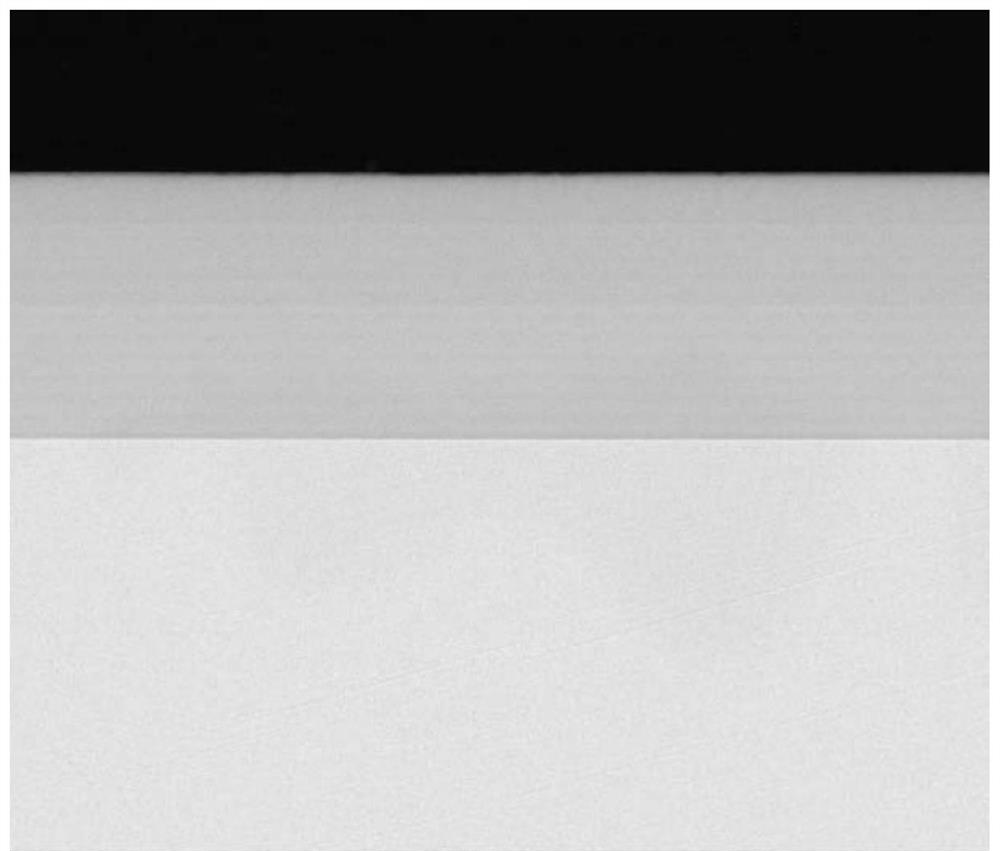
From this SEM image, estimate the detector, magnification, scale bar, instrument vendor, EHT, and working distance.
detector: A+B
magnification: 2.5 K X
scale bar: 50000 nm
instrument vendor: FEI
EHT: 25 kV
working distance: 10.5 mm